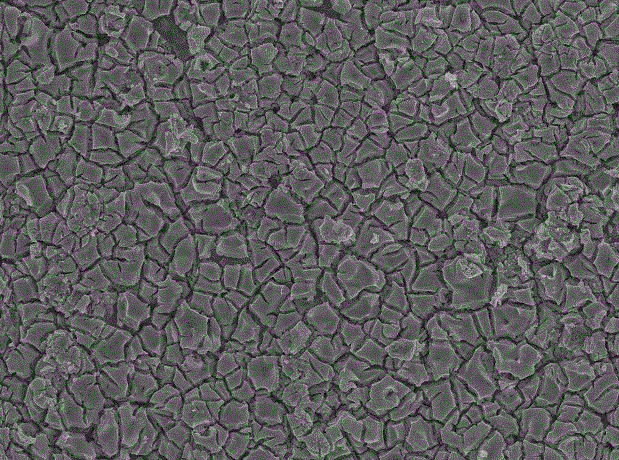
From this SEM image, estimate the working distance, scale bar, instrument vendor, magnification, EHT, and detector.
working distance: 13.9 mm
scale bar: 10000 nm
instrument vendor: JEOL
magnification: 1 K X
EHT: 15 kV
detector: SEI